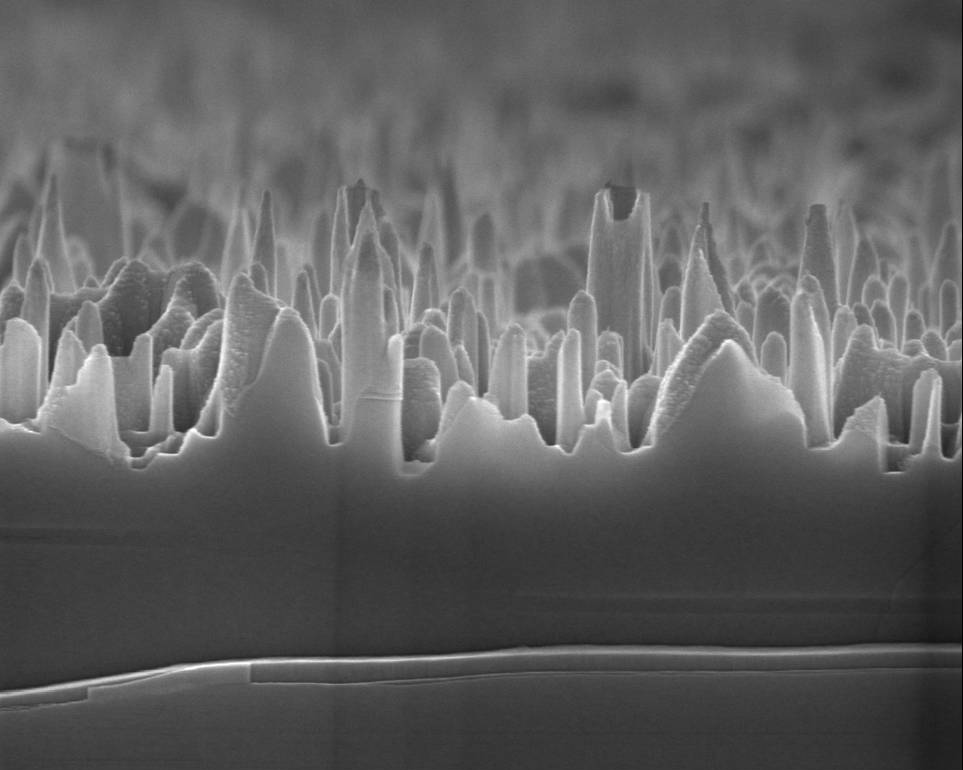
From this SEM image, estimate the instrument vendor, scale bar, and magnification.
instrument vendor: FEI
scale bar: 3000 nm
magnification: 80 K X